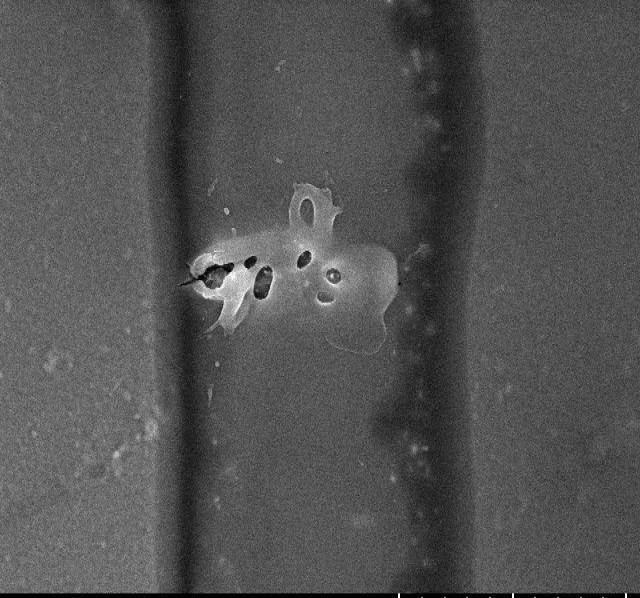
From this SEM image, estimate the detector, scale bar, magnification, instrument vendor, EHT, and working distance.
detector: SE(UL)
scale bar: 3000 nm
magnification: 15 K X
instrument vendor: Hitachi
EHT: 5 kV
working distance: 8.4 mm